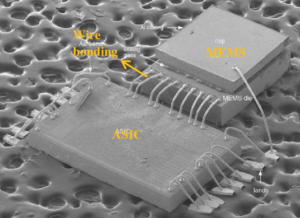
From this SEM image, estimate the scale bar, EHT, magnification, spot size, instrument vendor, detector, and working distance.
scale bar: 500000 nm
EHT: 10 kV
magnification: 0.035 K X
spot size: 3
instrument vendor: FEI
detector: SE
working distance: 14.5 mm